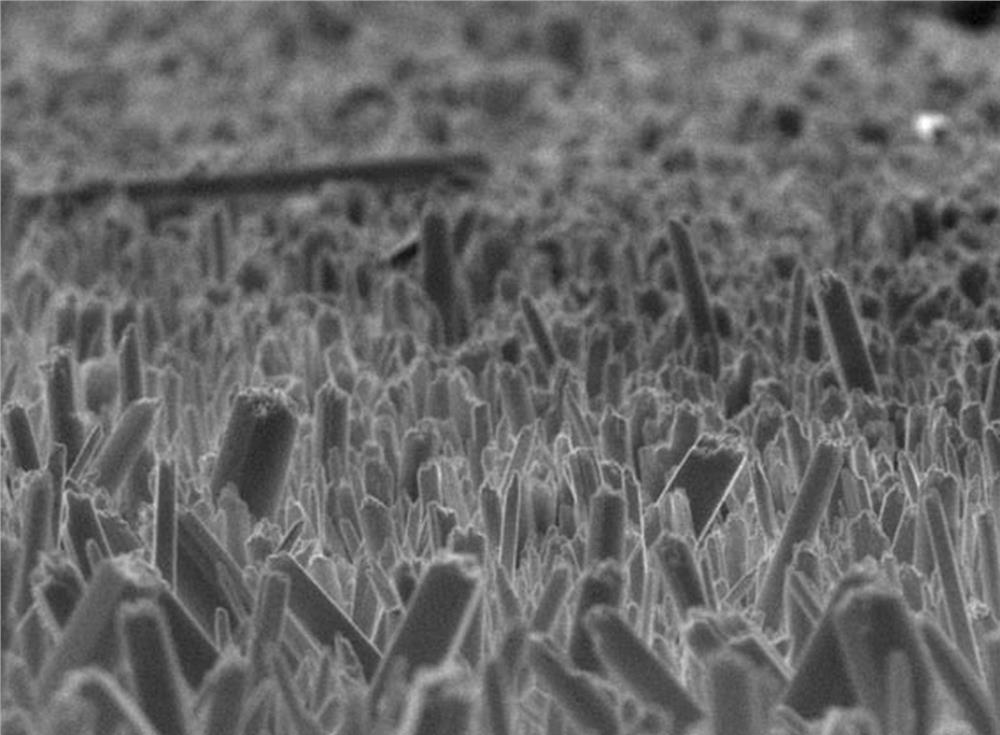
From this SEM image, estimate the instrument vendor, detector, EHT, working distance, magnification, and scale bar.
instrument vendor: JEOL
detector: SEI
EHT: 15 kV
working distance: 7.6 mm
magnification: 20 K X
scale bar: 1000 nm